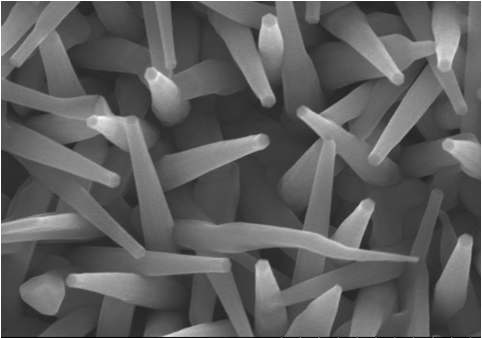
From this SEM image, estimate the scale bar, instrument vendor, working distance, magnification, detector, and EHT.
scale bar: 1000 nm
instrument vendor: Hitachi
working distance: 12.1 mm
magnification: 50 K X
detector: SE(M)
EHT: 15 kV